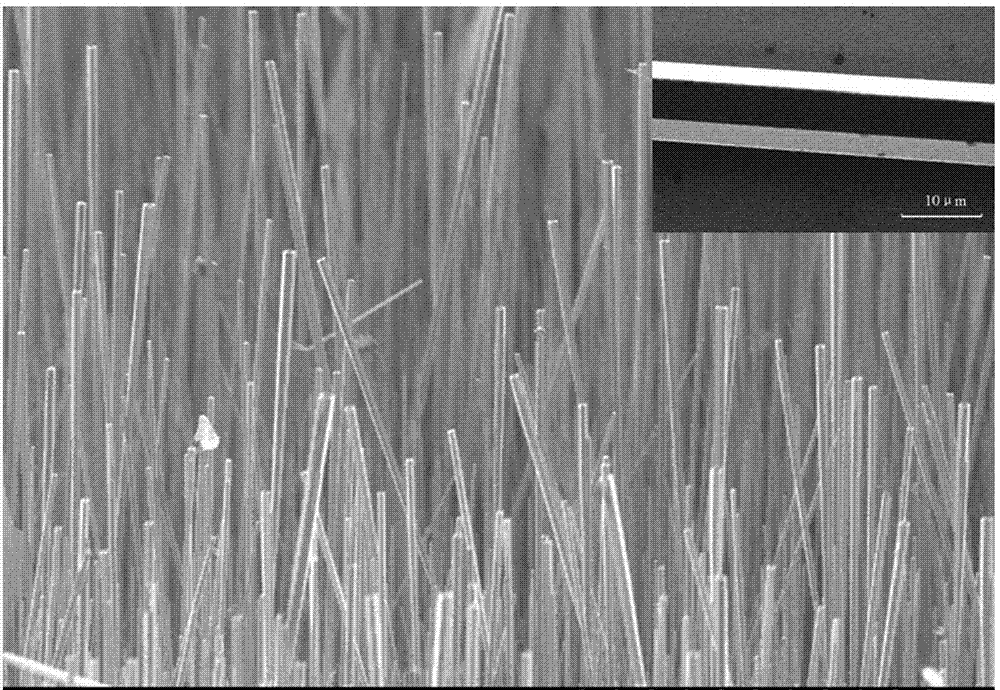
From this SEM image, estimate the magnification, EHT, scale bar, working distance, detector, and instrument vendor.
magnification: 0.09 K X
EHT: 10 kV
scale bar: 500000 nm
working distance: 26.9 mm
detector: SE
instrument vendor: Hitachi